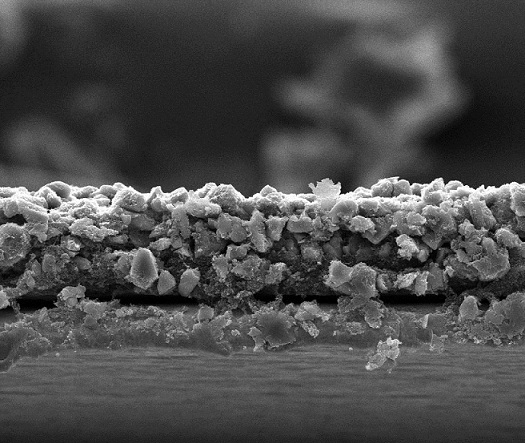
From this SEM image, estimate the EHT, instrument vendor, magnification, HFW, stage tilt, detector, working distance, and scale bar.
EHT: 20 kV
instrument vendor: FEI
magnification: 2 K X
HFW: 149 µm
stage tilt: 0°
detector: ETD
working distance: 9.6 mm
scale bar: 50000 nm